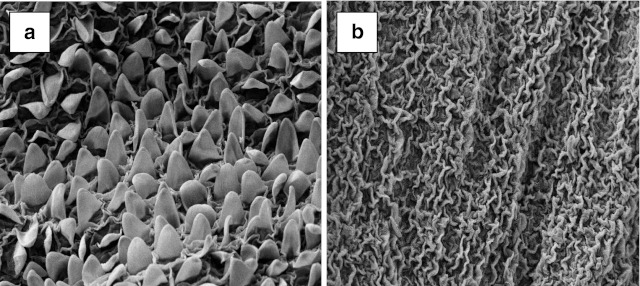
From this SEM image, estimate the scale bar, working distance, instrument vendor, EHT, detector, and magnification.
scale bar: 10000 nm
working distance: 11 mm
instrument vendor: Zeiss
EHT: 15 kV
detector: SE1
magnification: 1 K X